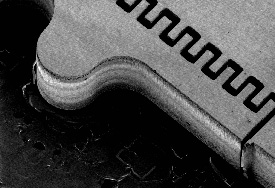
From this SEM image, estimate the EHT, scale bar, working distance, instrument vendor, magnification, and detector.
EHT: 1 kV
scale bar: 1000000 nm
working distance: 12.8 mm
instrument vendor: Hitachi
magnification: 0.05 K X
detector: SE(U)